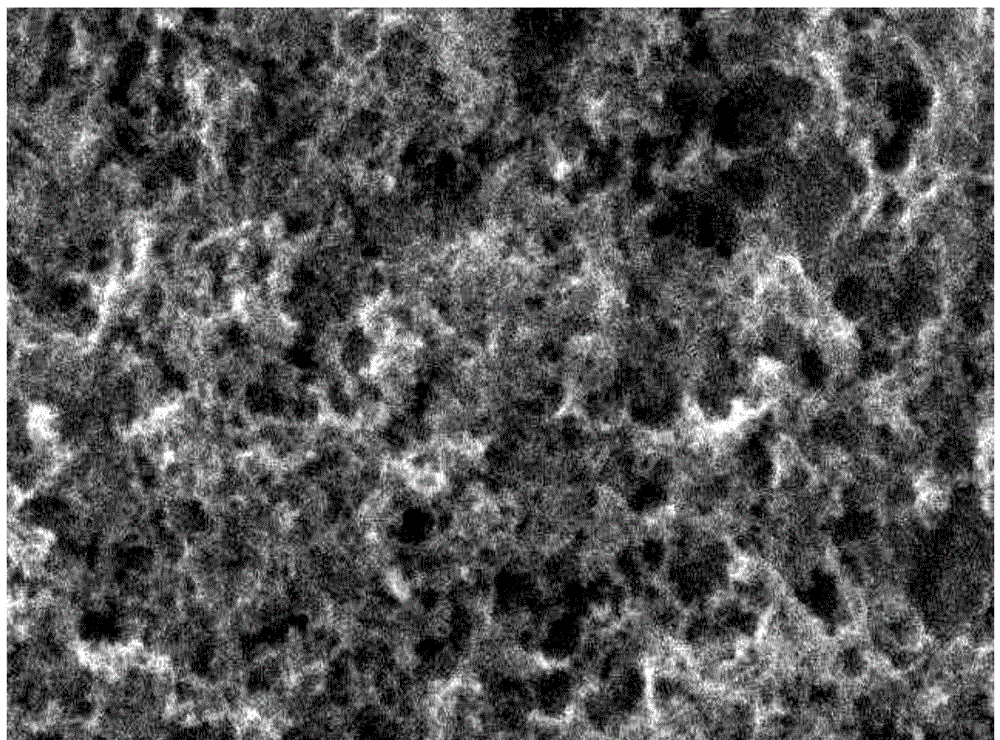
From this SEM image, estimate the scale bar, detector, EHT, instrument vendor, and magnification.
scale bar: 5000 nm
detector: SEI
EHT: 25 kV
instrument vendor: JEOL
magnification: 5 K X